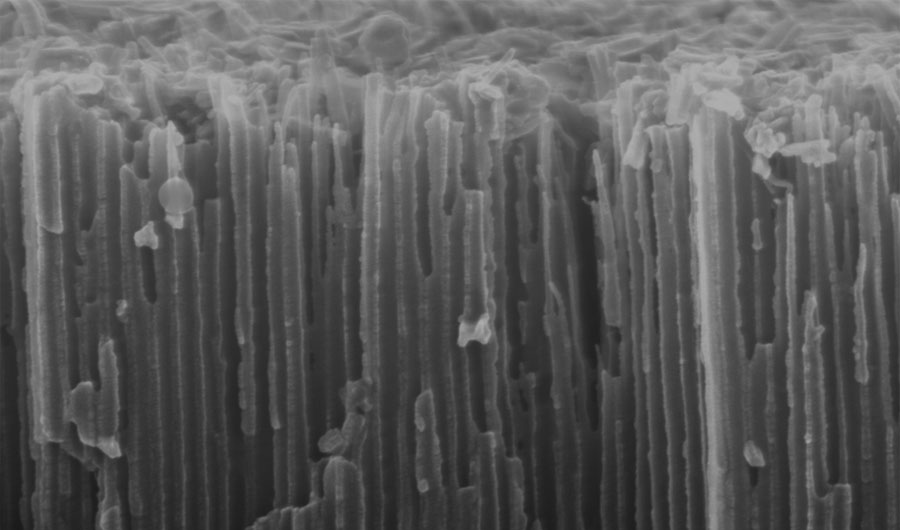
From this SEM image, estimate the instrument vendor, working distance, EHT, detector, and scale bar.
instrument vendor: Zeiss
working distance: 3 mm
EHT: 10 kV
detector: InLens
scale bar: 100 nm